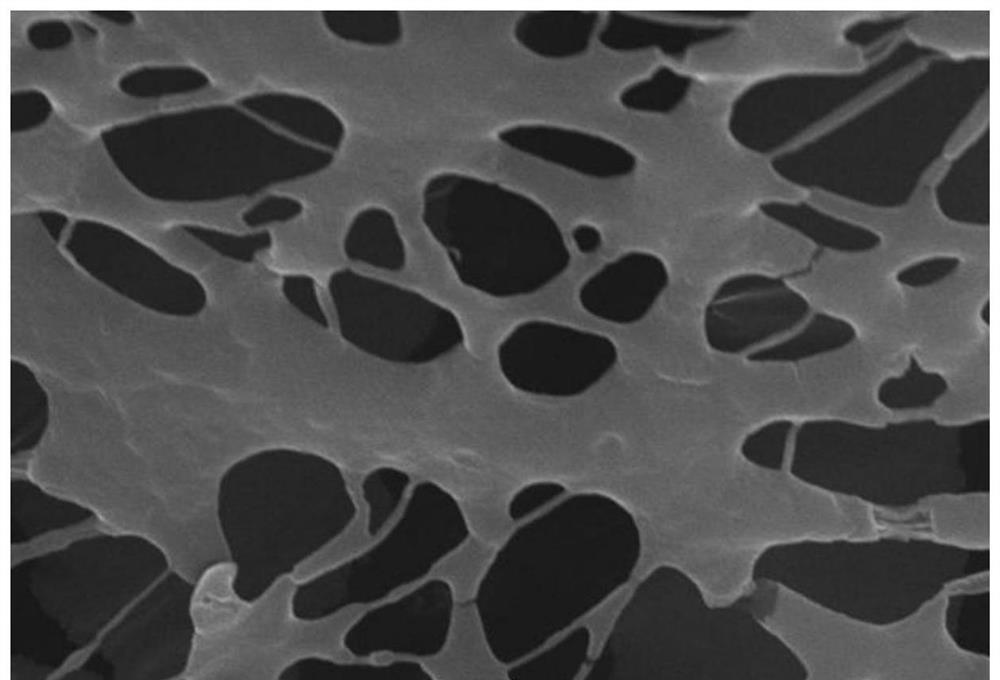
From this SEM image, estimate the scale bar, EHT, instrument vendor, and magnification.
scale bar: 1000 nm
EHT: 20 kV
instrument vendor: JEOL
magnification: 10 K X